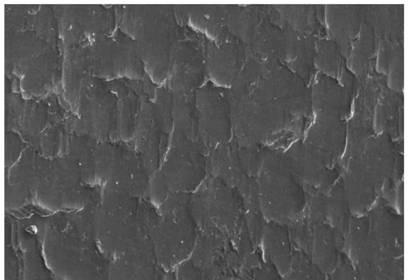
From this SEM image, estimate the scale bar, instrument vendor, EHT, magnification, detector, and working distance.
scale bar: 20000 nm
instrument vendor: Zeiss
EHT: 20 kV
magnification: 0.5 K X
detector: SE1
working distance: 12 mm